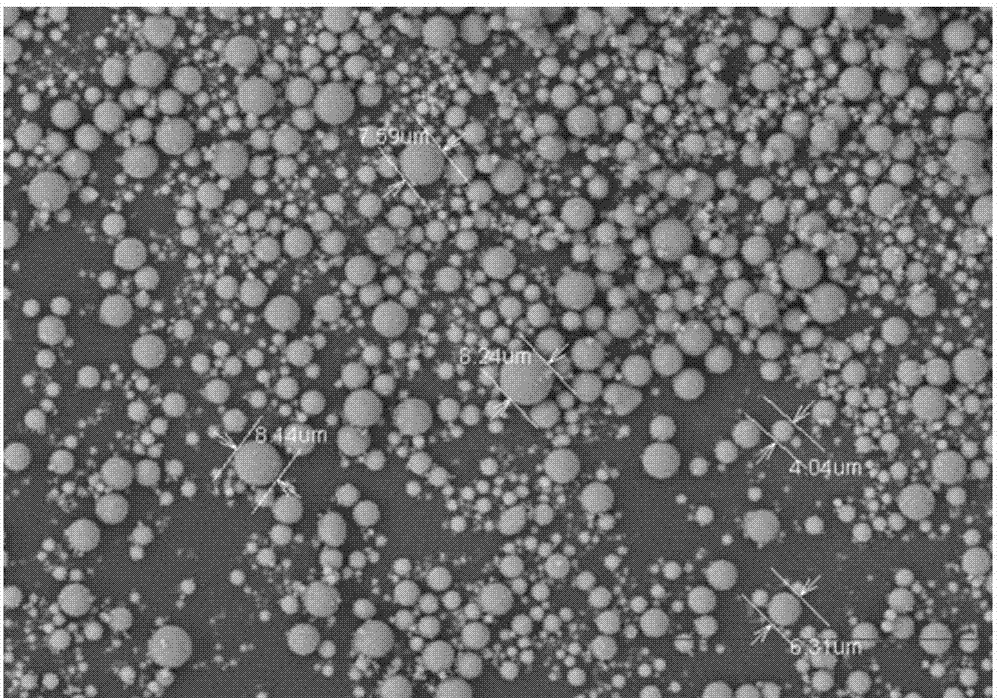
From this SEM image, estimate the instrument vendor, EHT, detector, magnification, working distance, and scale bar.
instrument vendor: Hitachi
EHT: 15 kV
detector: BSE3D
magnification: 0.8 K X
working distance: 10.3 mm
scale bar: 50000 nm